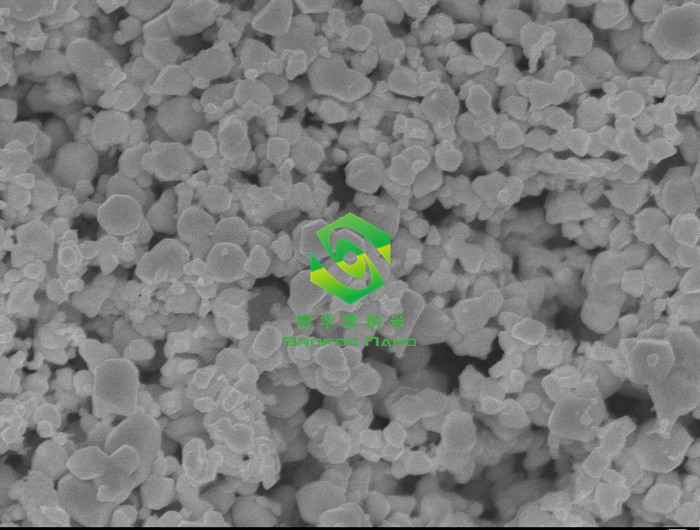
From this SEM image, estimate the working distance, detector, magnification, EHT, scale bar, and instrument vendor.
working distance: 8 mm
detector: SEI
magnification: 30 K X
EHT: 10 kV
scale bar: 100 nm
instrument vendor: JEOL